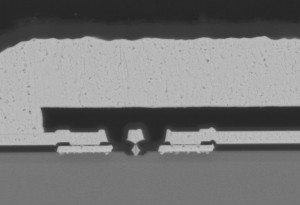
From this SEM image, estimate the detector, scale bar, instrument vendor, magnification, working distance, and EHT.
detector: BSECOMP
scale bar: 5000 nm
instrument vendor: JEOL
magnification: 8 K X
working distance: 10.6 mm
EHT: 15 kV